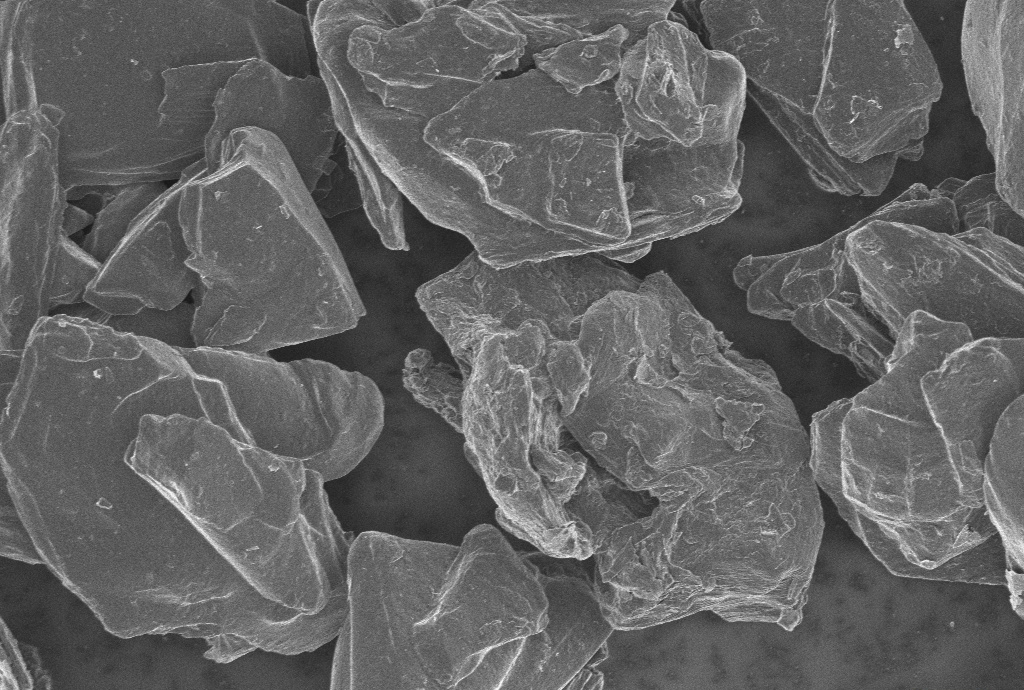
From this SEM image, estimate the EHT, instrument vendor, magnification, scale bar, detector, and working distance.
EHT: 1 kV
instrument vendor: Zeiss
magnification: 3 K X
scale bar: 2000 nm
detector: InLens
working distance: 5.7 mm